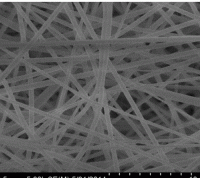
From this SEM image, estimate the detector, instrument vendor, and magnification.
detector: SE(M)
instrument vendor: Hitachi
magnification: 5 K X